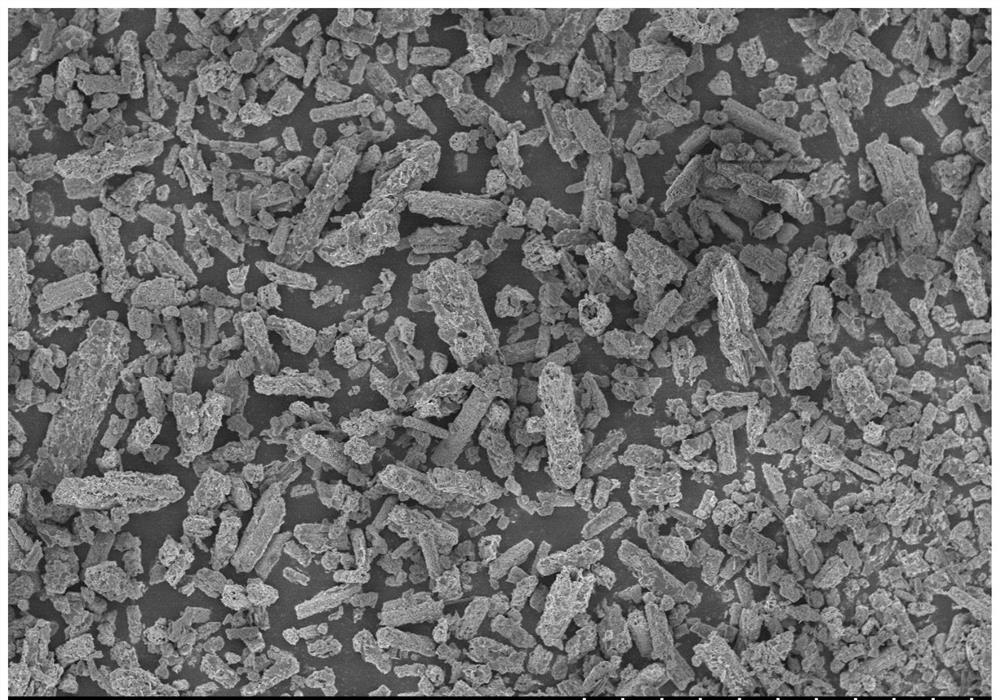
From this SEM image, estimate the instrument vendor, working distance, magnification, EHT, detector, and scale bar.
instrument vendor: Hitachi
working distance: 7.1 mm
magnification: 1 K X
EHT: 3 kV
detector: SE(U)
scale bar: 50000 nm